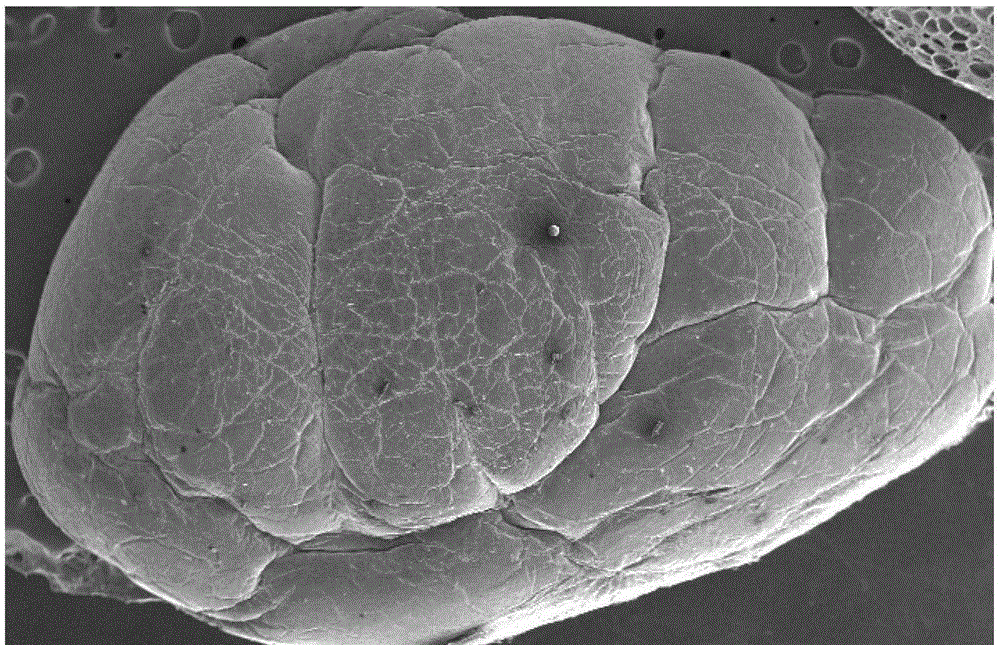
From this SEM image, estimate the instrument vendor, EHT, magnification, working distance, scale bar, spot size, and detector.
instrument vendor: FEI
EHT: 10 kV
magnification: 0.019 K X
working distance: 19.9 mm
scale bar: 1000000 nm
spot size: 3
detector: SE